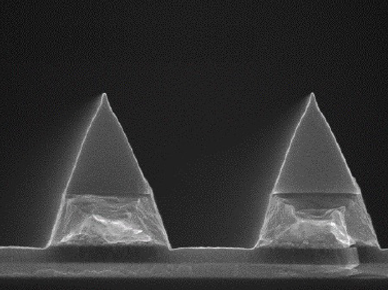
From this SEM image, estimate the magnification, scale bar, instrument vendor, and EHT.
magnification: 30 K X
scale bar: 1000 nm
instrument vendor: JEOL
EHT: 10 kV